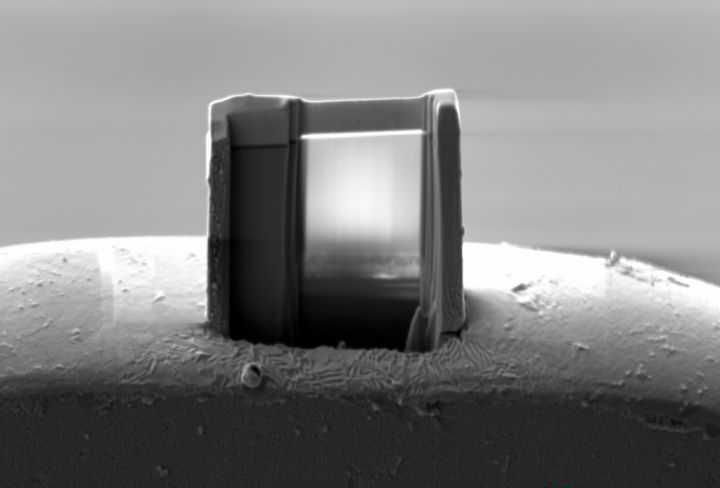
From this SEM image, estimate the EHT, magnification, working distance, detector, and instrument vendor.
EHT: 5 kV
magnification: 3.92 K X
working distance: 5.2 mm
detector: SE2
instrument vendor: Zeiss